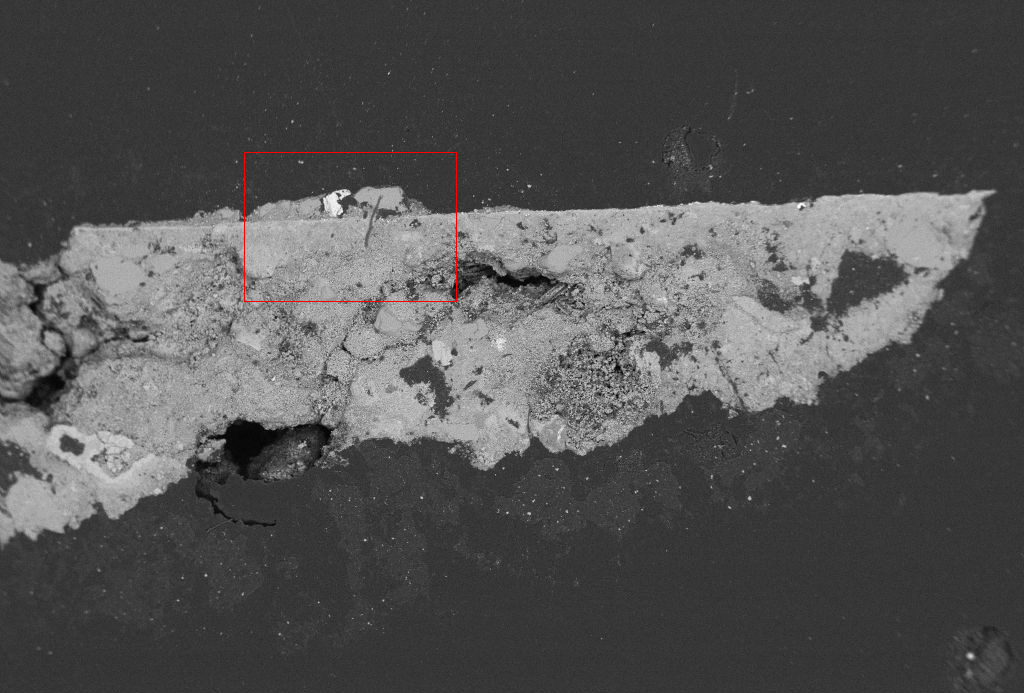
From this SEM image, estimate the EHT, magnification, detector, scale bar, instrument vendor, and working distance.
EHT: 20 kV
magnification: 0.081 K X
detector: BSD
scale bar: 200000 nm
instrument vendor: Zeiss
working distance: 21 mm